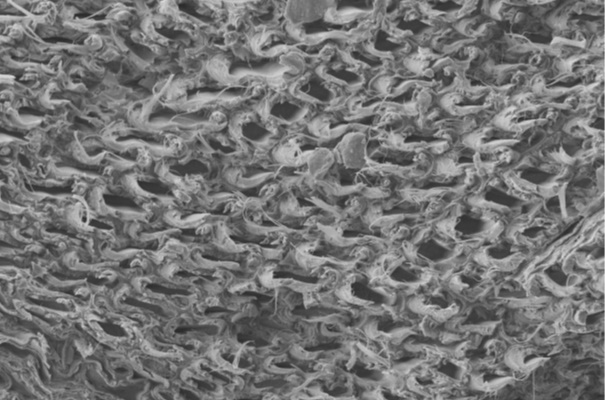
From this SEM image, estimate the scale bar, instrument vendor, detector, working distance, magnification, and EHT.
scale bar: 20000 nm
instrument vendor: Zeiss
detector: SE2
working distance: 19 mm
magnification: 1 K X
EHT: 10 kV